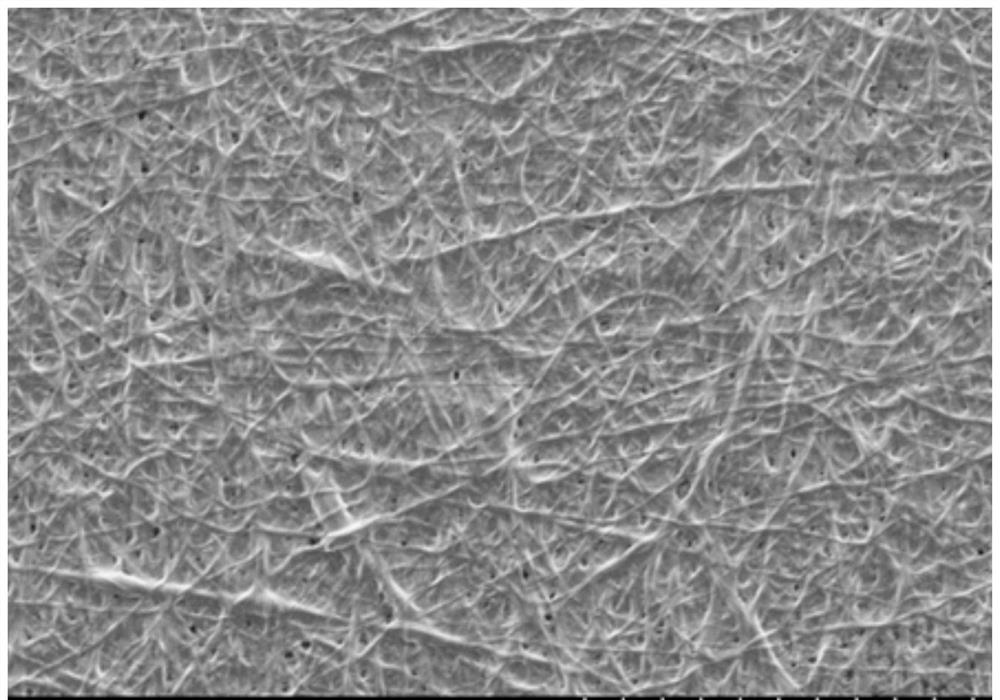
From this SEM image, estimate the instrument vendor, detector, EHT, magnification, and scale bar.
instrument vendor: Hitachi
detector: SE(M)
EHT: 4 kV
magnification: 0.5 K X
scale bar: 100000 nm